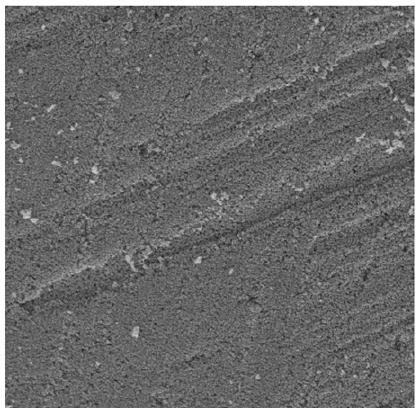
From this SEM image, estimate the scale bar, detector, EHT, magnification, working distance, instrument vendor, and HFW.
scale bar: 20000 nm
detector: SE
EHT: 30 kV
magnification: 2 K X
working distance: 11.01 mm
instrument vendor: Tescan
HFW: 142 µm